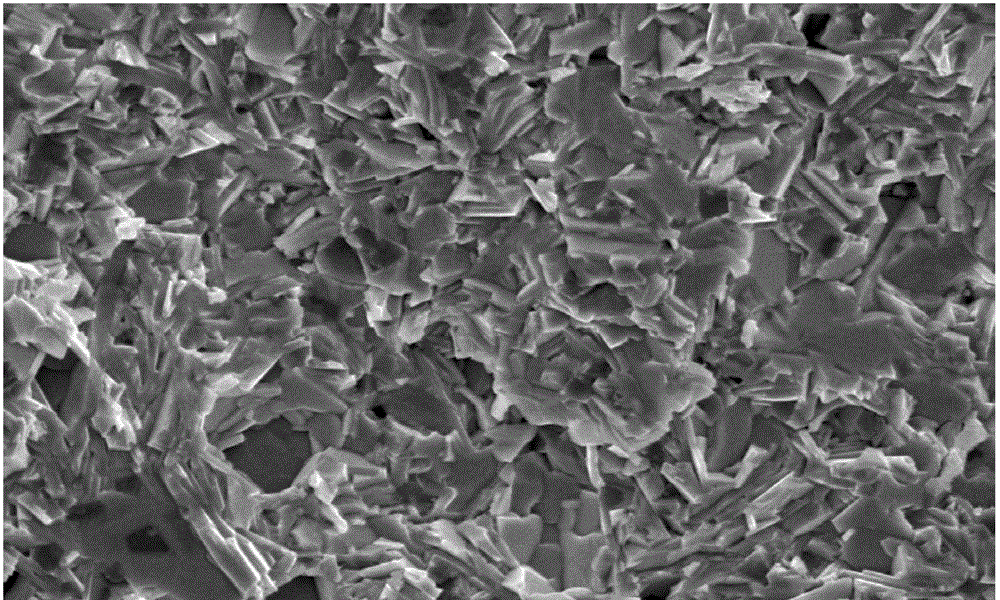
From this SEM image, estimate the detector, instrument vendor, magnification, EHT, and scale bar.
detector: SEI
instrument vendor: JEOL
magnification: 2 K X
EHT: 20 kV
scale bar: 10000 nm